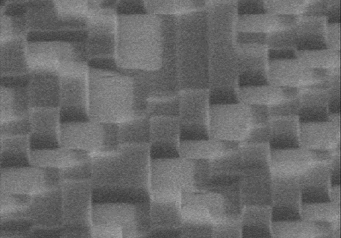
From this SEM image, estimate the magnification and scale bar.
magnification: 30 K X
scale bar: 1000 nm